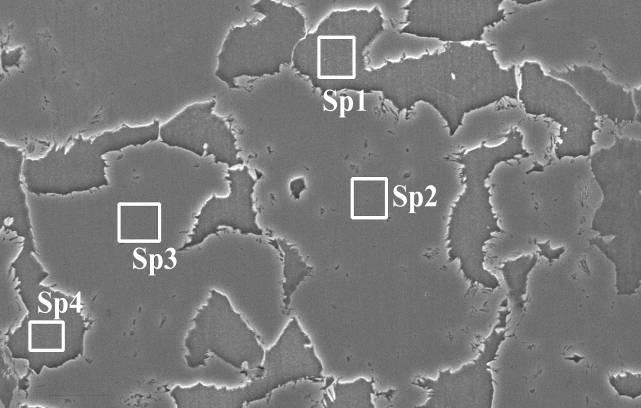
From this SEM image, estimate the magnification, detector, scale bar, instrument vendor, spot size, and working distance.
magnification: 1.5 K X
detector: SE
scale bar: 20000 nm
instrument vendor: FEI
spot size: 5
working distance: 10.8 mm